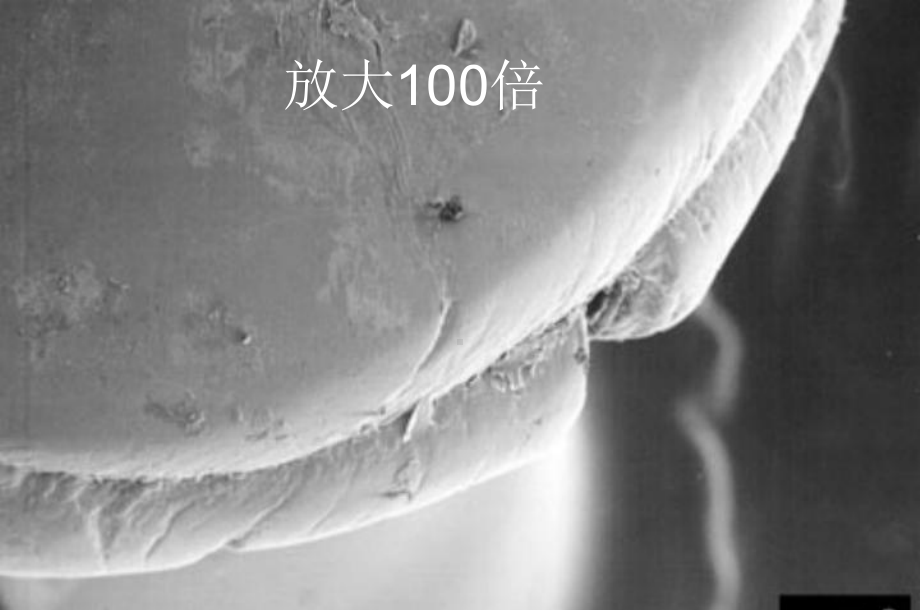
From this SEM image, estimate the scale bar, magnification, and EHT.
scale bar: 100000 nm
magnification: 0.1 K X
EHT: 10 kV